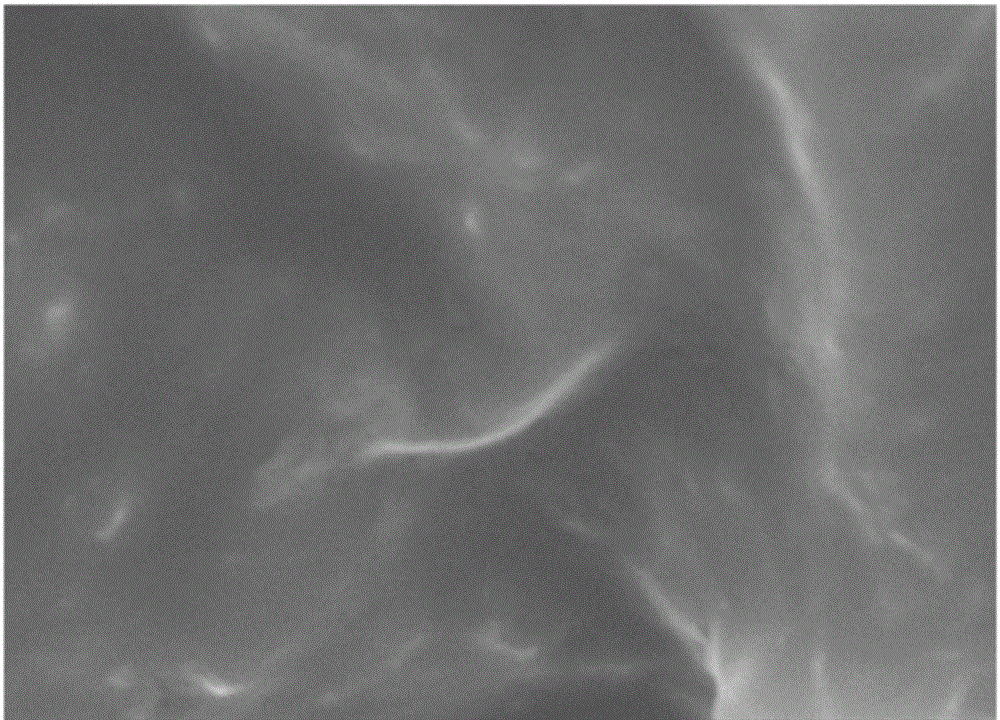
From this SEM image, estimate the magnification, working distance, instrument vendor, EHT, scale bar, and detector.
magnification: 60 K X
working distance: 8.8 mm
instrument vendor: Hitachi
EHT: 5 kV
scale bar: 500 nm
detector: SE(M)